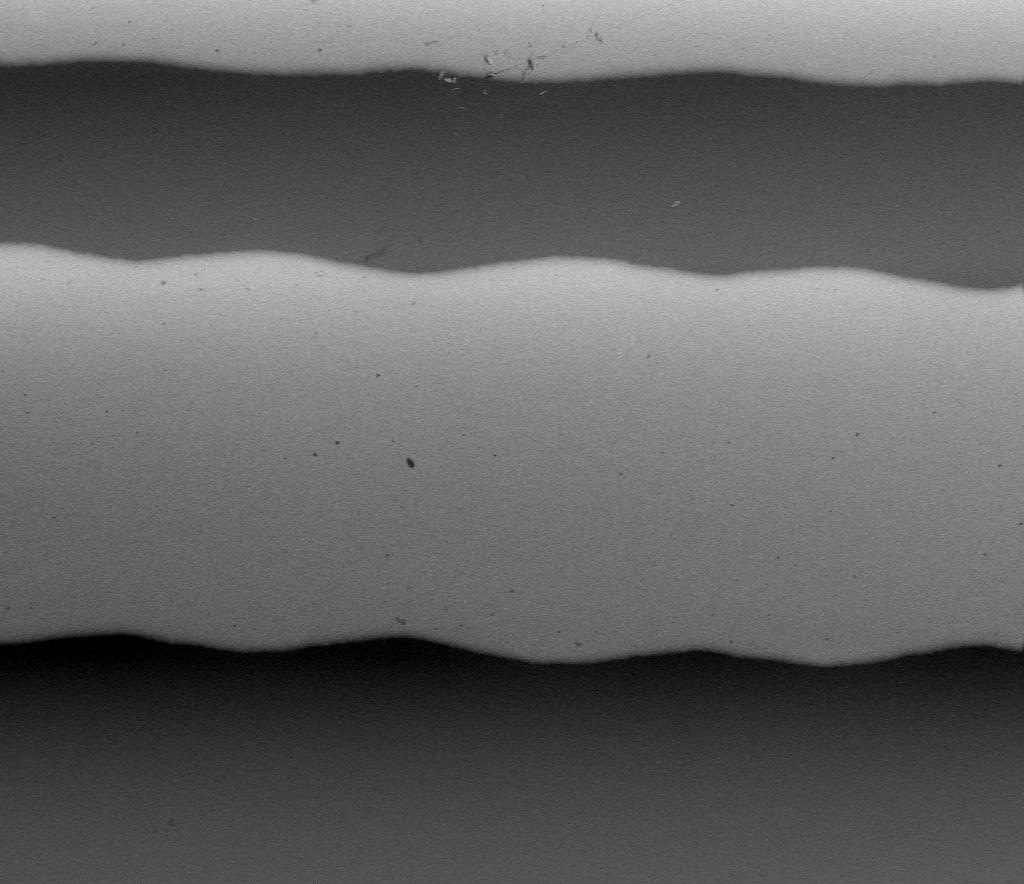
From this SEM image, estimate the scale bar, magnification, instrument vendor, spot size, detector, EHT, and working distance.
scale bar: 200000 nm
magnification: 0.5 K X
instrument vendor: FEI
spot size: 5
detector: SSD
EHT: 10 kV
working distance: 10.7 mm